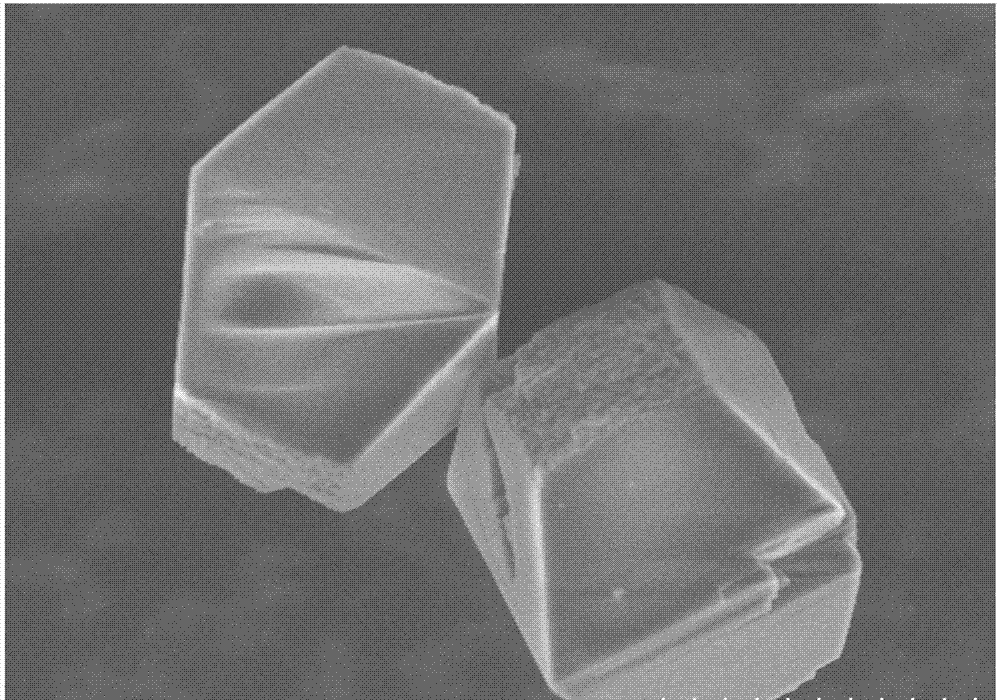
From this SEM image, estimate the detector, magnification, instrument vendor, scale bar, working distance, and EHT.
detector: SE(M)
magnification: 4 K X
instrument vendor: Hitachi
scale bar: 10000 nm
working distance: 7.8 mm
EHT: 5 kV